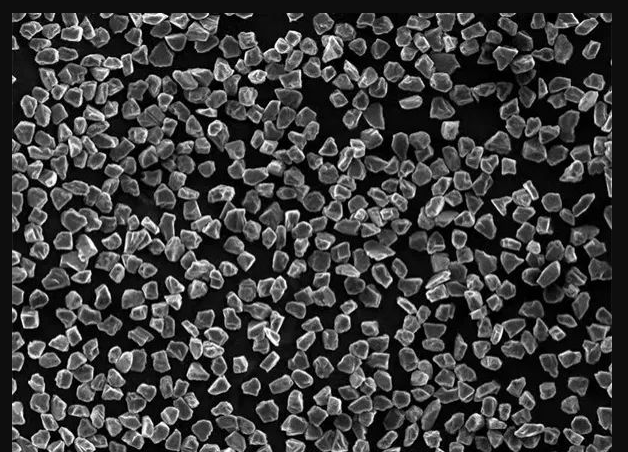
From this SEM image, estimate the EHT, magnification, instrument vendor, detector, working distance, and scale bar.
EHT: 15 kV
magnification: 0.5 K X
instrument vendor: JEOL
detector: SEI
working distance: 10 mm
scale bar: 10000 nm